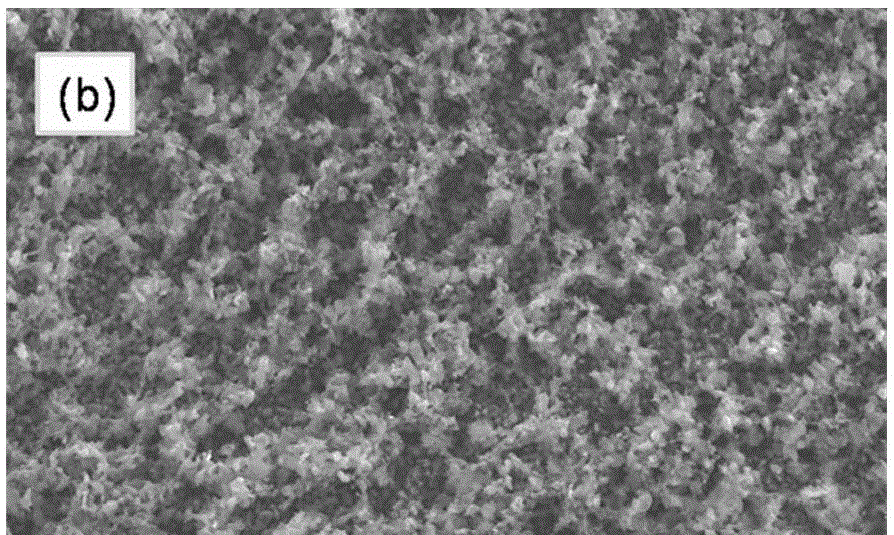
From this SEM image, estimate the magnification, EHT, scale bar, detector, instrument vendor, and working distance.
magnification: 1 K X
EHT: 15 kV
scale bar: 50000 nm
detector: SE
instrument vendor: Hitachi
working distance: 9.8 mm